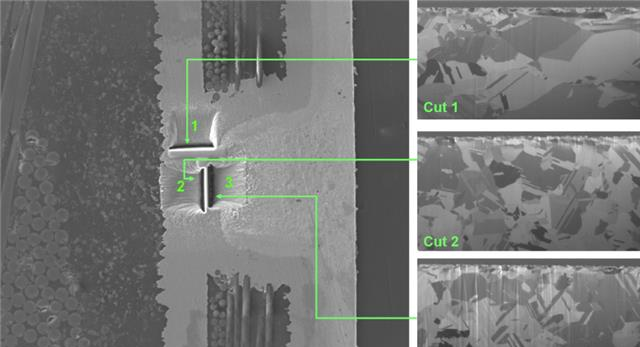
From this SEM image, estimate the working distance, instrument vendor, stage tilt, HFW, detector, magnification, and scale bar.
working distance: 15.8 mm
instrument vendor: FEI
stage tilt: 0°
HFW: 298 µm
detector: ETD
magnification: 1 K X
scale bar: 50000 nm